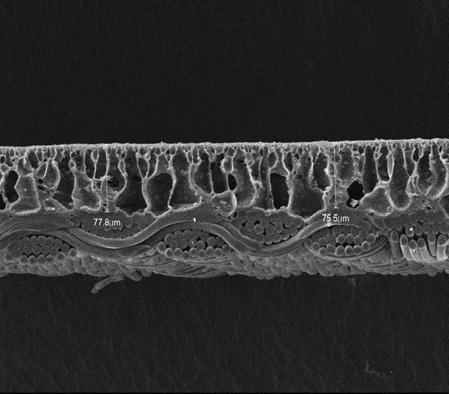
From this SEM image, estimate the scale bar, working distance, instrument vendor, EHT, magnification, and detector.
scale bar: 100000 nm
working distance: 8 mm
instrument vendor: JEOL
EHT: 15 kV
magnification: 0.2 K X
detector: LEI